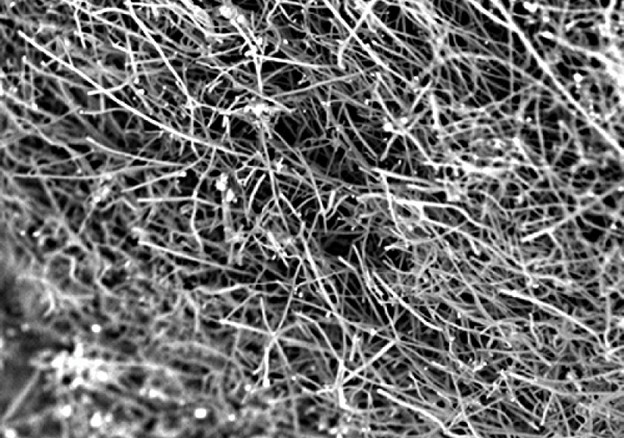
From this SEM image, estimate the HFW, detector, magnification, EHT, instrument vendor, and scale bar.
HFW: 67.2 µm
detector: BSD Full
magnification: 4 K X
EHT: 10 kV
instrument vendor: other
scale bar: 20000 nm